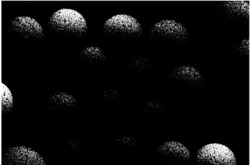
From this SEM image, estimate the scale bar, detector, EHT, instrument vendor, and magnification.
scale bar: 5000 nm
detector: SEI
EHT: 20 kV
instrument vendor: JEOL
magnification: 5 K X